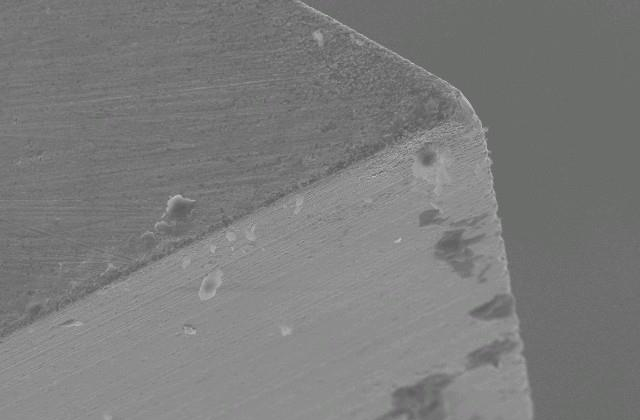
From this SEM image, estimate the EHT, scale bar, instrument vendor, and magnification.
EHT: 20 kV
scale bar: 50000 nm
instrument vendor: JEOL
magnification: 0.5 K X